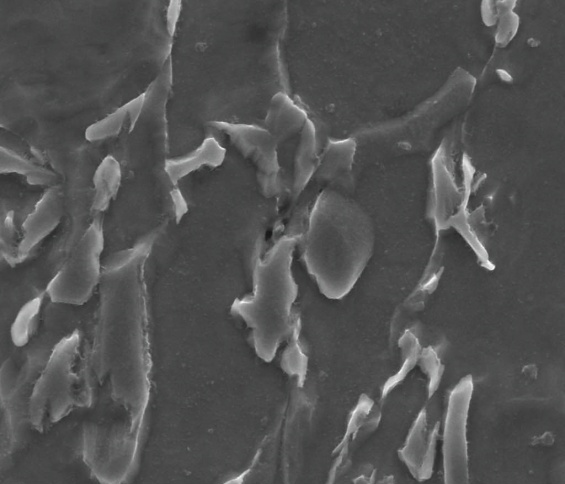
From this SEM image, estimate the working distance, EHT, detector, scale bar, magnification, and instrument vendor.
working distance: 9.9 mm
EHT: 15 kV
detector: SE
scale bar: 5000 nm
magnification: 20 K X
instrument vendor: FEI